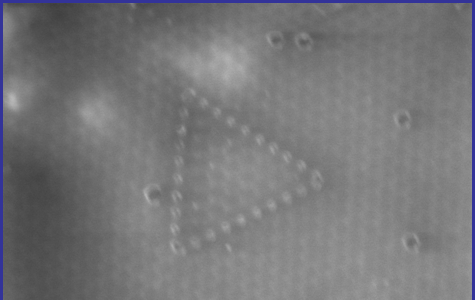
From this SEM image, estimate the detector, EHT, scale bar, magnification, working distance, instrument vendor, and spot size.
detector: GSE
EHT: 10 kV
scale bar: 2000 nm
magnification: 12.8 K X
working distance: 9.2 mm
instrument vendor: FEI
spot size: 4